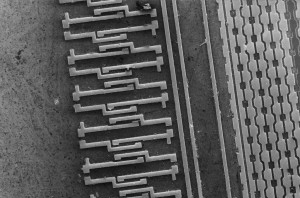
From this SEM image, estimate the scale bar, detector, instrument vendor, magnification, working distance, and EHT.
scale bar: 10000 nm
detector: SE1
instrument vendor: Zeiss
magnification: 0.562 K X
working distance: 11 mm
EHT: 4 kV